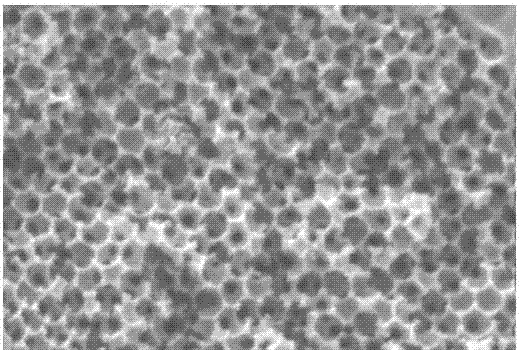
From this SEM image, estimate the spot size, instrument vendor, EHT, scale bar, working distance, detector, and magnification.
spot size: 3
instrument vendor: FEI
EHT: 20 kV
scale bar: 1000 nm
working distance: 10.7 mm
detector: SE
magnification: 20 K X